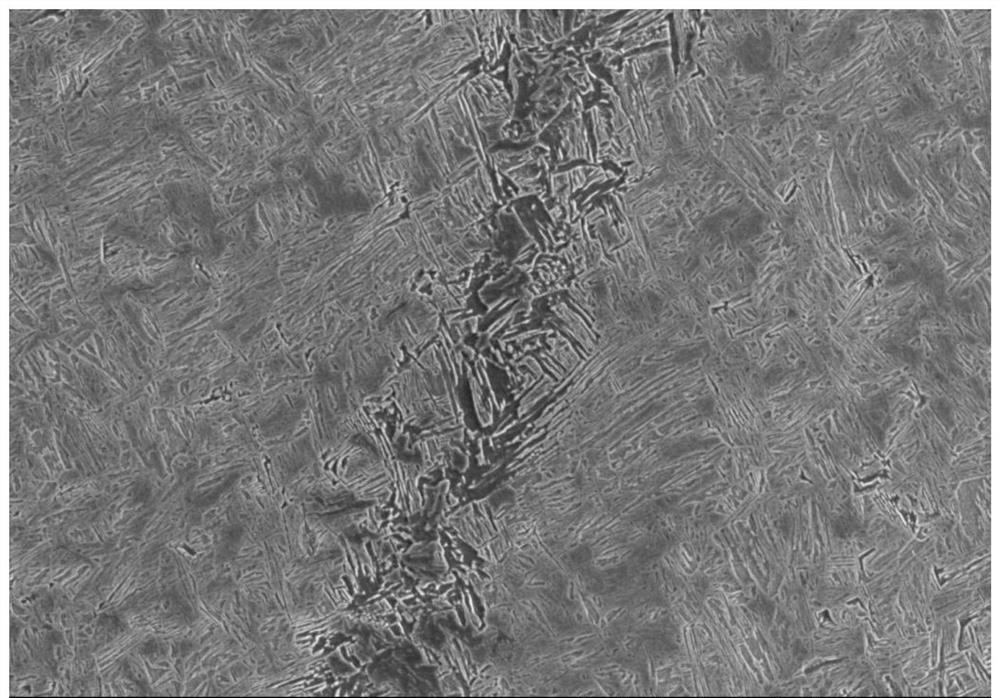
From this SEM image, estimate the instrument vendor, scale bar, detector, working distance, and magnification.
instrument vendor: Hitachi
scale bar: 50000 nm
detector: SE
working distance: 9.5 mm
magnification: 1 K X